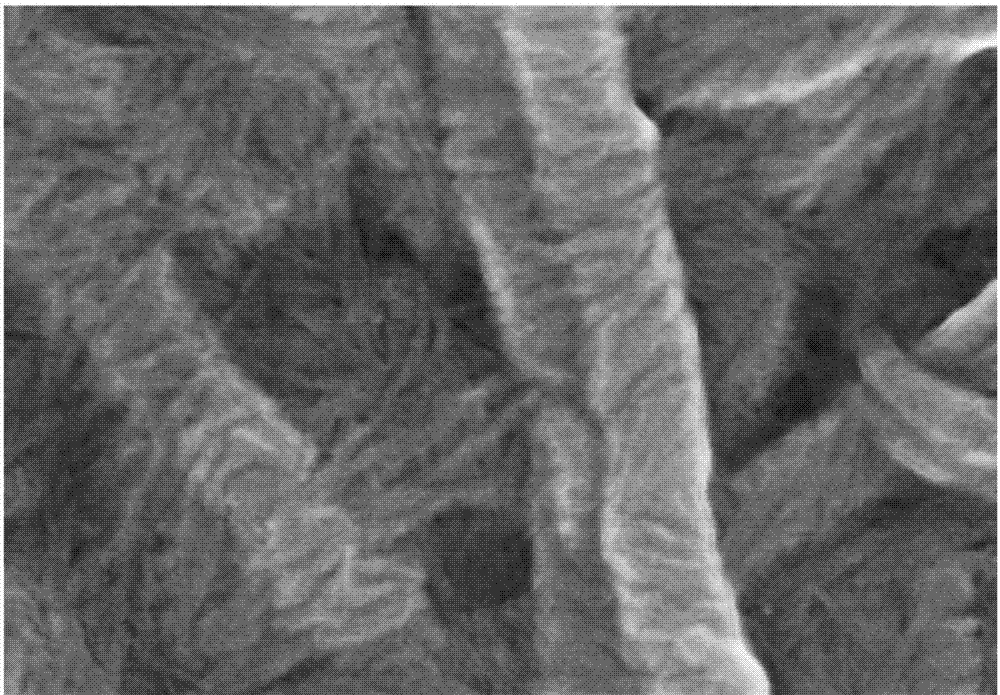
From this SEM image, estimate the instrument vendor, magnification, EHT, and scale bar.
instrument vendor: Hitachi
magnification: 100 K X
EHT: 5 kV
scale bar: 500 nm